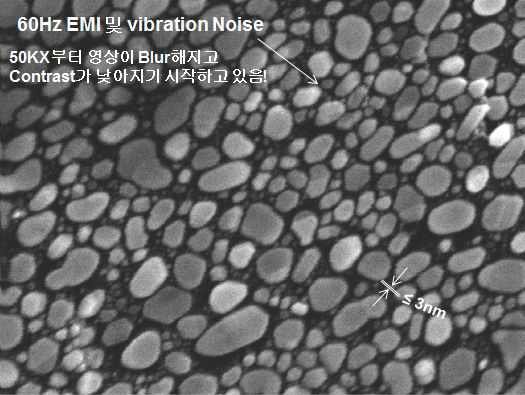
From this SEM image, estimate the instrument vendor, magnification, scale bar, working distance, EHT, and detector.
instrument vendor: other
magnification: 100 K X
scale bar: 500 nm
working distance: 8.8 mm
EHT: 20 kV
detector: SEI